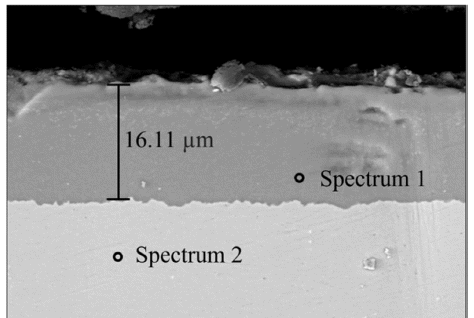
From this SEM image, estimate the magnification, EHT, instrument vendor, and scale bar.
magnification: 2 K X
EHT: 20 kV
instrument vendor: JEOL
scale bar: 10000 nm